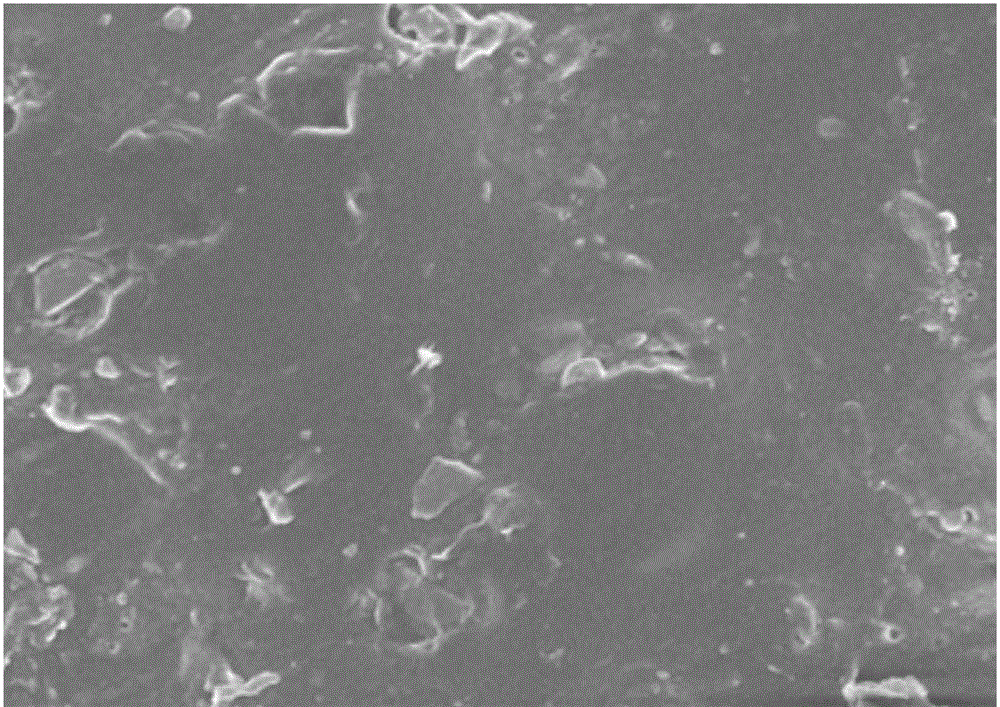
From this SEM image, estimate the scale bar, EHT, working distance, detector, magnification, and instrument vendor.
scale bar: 1000 nm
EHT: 5 kV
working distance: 9.7 mm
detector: SEI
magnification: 3 K X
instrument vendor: JEOL